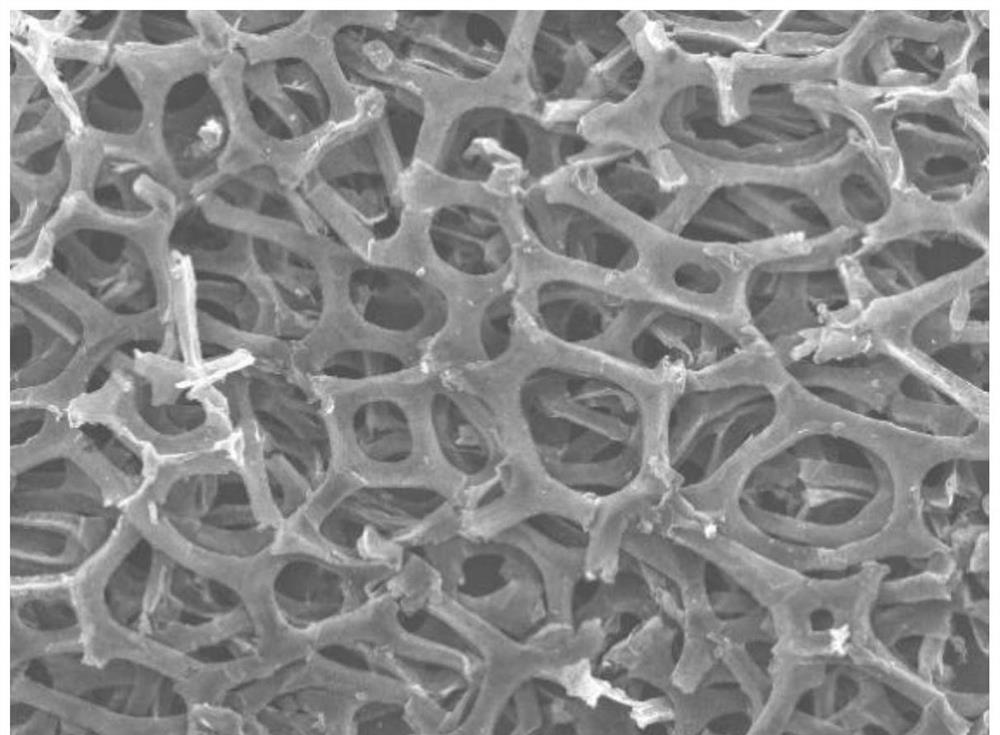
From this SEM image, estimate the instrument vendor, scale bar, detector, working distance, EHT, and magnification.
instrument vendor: JEOL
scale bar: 100000 nm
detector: SEI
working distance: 8 mm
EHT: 15 kV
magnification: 0.05 K X